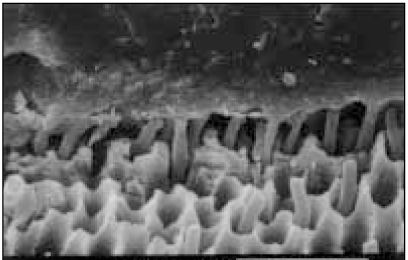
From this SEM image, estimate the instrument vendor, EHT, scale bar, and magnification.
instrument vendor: JEOL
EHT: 20 kV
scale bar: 20000 nm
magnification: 2 K X